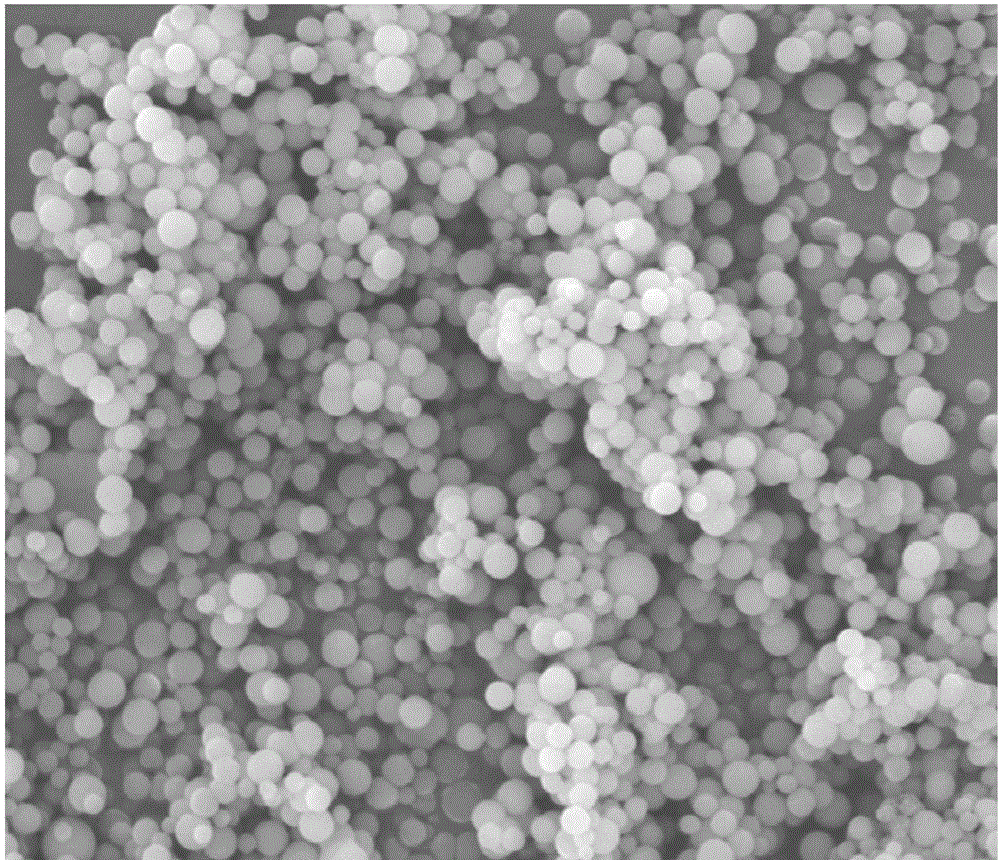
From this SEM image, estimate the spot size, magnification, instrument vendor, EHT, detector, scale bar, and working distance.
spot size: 3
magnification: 5 K X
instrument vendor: FEI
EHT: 20 kV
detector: ETD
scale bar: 10000 nm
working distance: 13.6 mm